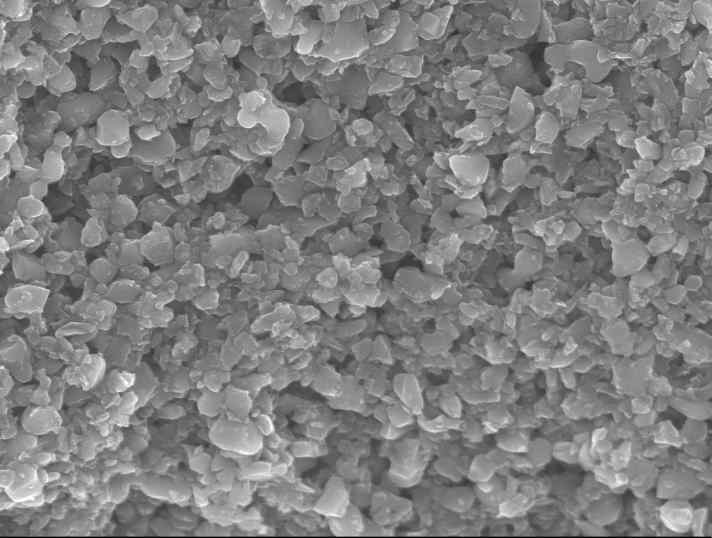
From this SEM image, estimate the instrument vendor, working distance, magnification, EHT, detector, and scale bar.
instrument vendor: JEOL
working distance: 8.2 mm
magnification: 10 K X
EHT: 15 kV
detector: SEI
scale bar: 1000 nm